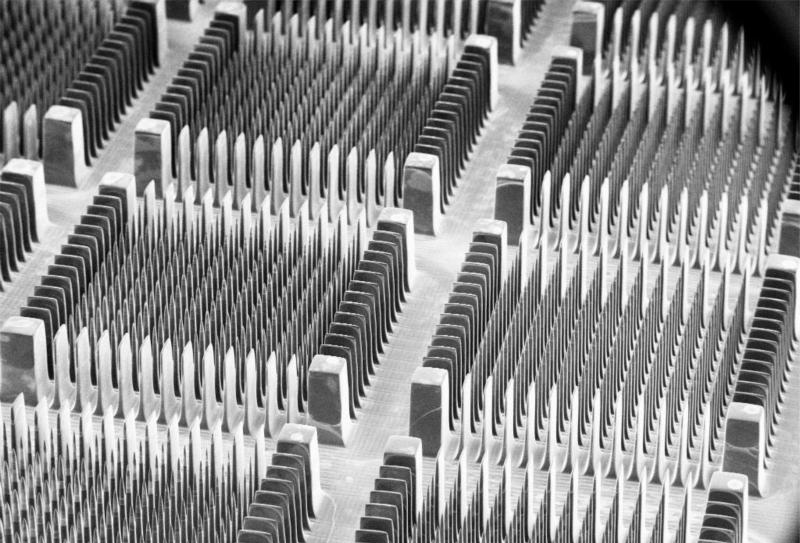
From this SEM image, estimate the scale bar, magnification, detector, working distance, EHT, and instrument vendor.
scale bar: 1000000 nm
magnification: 0.022 K X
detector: SE1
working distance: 50 mm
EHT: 10 kV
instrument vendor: Zeiss